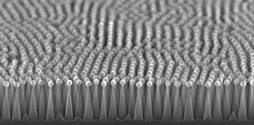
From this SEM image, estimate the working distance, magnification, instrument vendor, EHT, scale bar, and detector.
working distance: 5.7 mm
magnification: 100 K X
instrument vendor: Hitachi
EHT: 20 kV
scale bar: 500 nm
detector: SE(U,LA0)